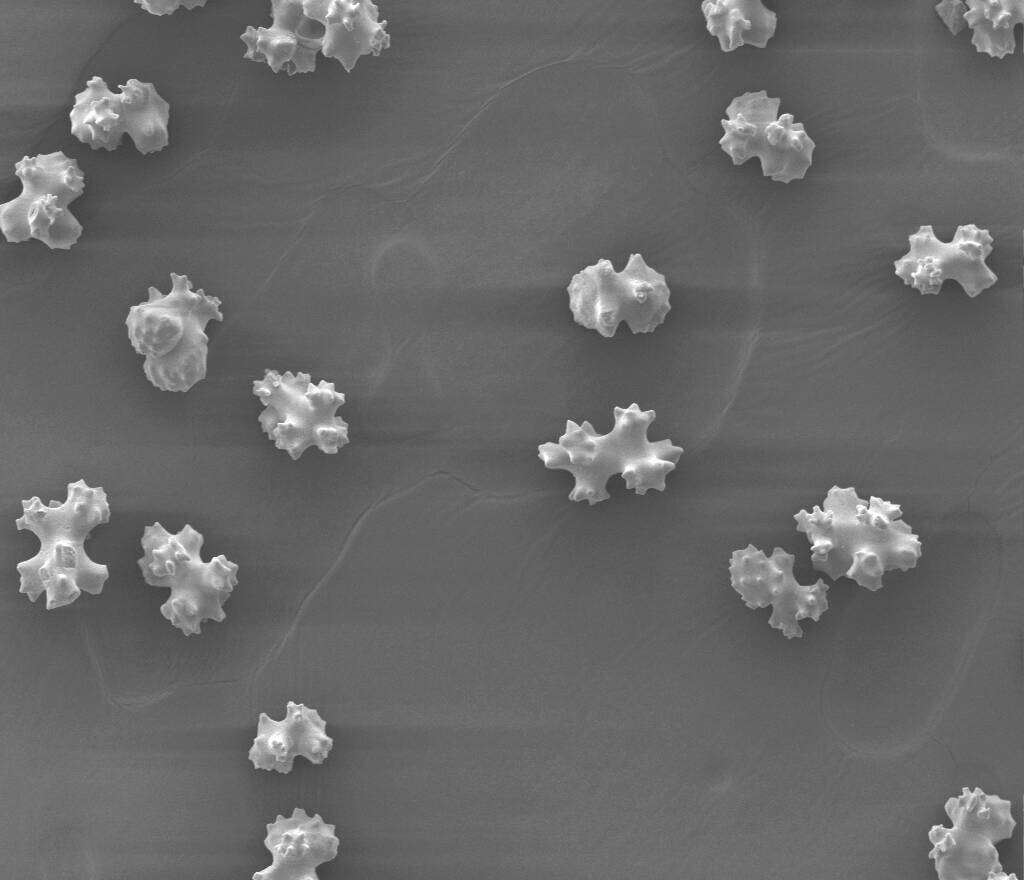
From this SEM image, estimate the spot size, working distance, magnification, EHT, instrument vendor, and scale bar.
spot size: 4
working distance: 12 mm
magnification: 0.4 K X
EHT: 12.5 kV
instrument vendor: FEI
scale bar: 400000 nm